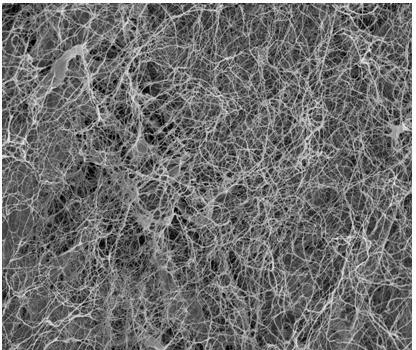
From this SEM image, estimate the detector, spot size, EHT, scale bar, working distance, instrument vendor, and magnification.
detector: ETD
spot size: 5.5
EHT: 12.5 kV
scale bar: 100000 nm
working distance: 9.8 mm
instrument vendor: FEI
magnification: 0.3 K X